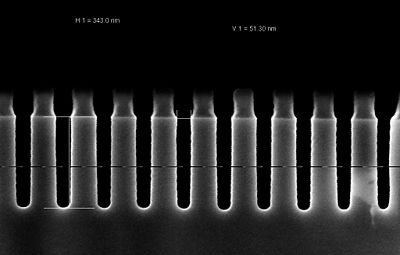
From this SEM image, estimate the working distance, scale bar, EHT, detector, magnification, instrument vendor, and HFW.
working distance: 3.2 mm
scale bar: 100 nm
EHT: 2 kV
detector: InLens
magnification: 200 K X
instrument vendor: Zeiss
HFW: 1.501 µm